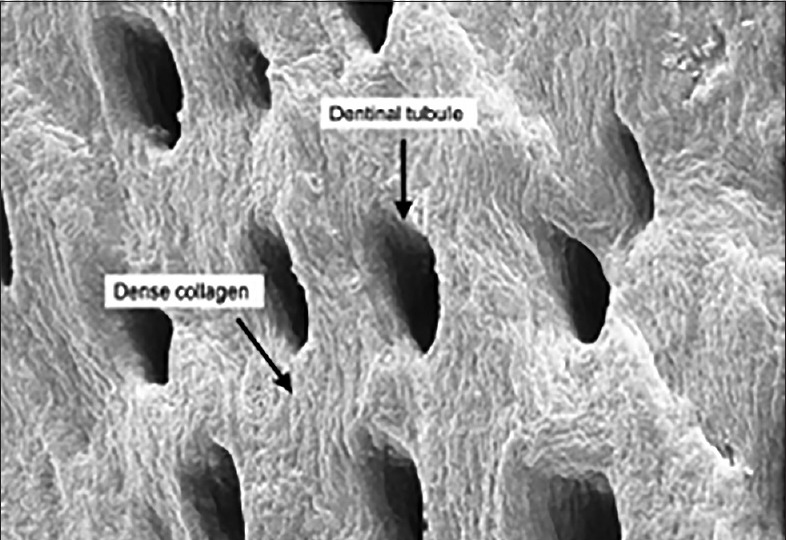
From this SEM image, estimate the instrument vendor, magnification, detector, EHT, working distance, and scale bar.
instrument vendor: Hitachi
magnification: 10 K X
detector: SE(M)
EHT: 3 kV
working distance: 21.7 mm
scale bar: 5000 nm